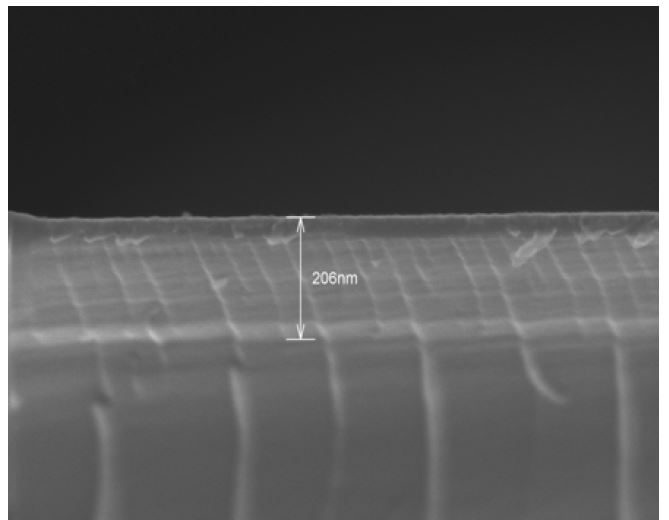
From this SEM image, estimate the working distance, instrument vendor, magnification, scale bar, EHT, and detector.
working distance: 7.4 mm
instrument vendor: Hitachi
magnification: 100 K X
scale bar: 500 nm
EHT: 10 kV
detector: SE(UL)